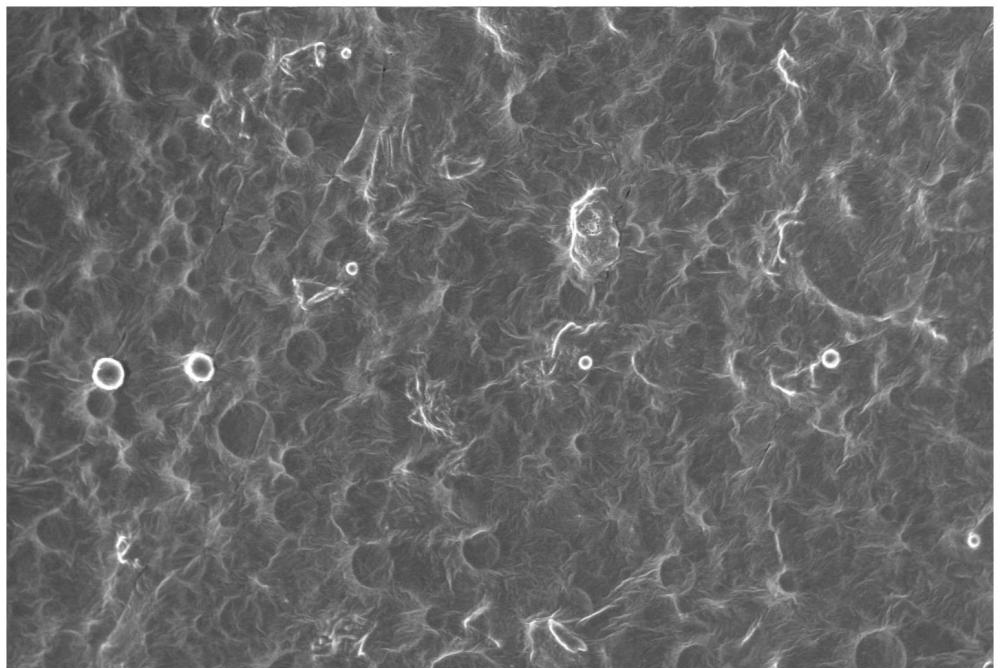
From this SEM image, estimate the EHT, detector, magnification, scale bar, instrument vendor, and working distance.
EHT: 10 kV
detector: InLens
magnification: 0.3 K X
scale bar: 20000 nm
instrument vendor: Zeiss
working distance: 4.7 mm